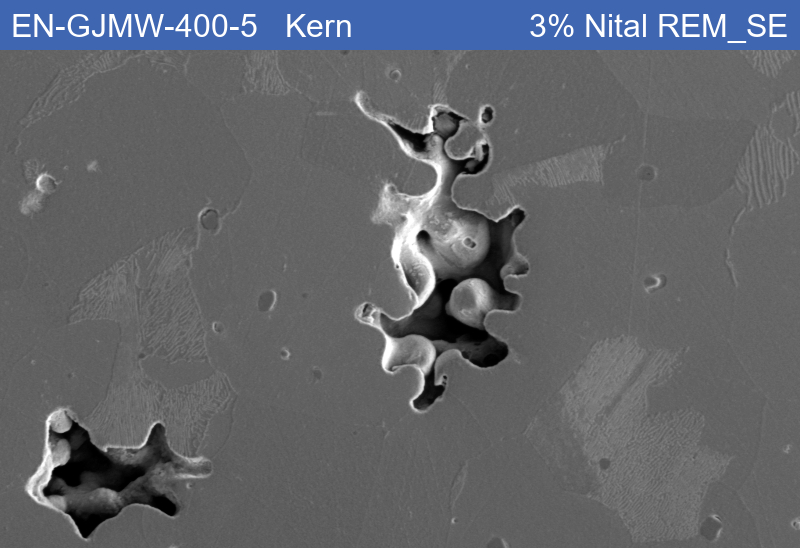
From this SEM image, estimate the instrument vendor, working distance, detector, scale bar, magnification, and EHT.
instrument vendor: Zeiss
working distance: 8.52 mm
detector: SE1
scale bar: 20000 nm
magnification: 0.5 K X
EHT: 15 kV